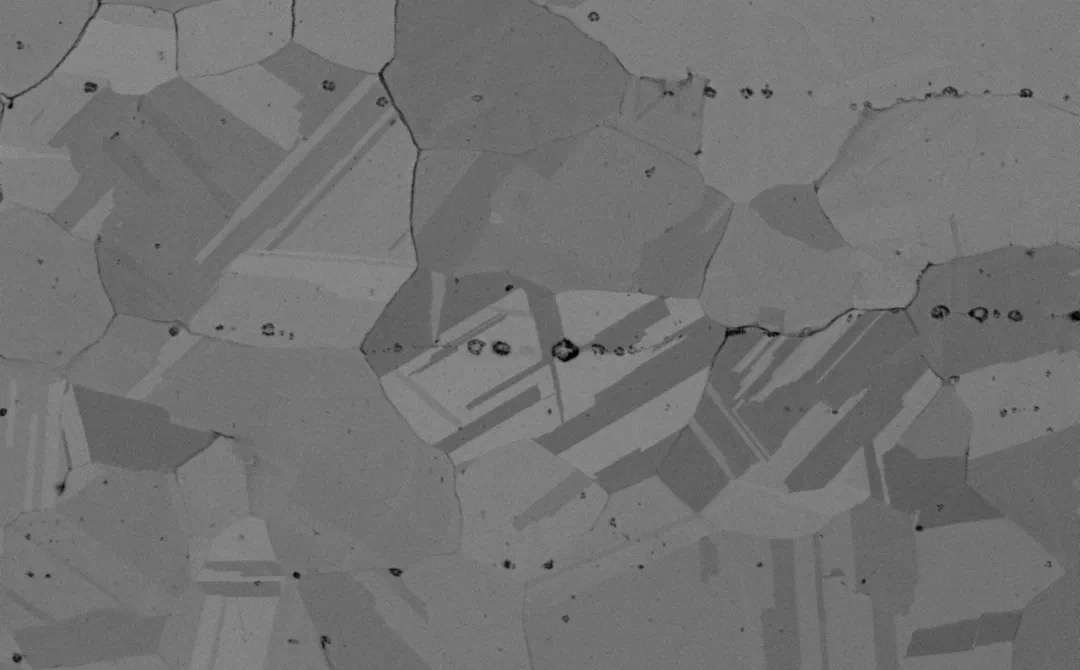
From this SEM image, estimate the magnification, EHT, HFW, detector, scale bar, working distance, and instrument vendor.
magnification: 5 K X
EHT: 10 kV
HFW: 25.4 µm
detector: BSD Full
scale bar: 8000 nm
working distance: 11.586 mm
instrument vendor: Thermo